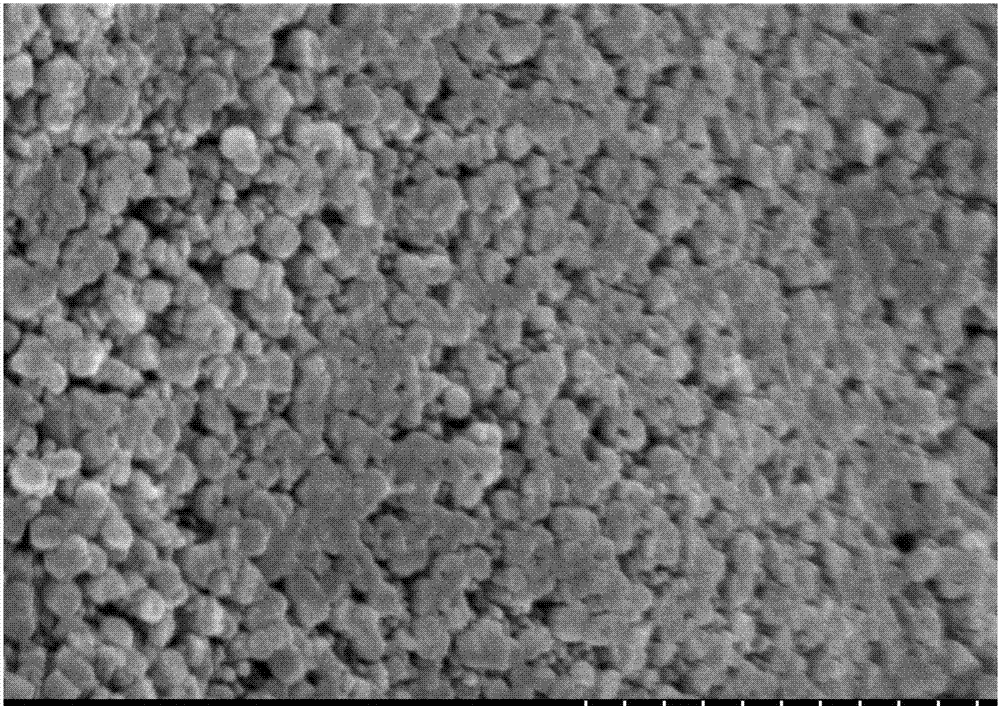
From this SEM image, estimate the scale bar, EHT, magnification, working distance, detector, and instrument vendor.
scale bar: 1000 nm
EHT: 3 kV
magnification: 50 K X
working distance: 9.2 mm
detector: SE(M)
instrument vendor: Hitachi